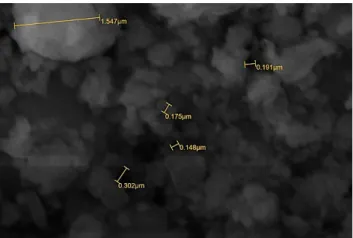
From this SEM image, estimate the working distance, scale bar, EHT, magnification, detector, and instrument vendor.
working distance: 19 mm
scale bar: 1000 nm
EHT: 20 kV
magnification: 20 K X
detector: SEI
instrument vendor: JEOL